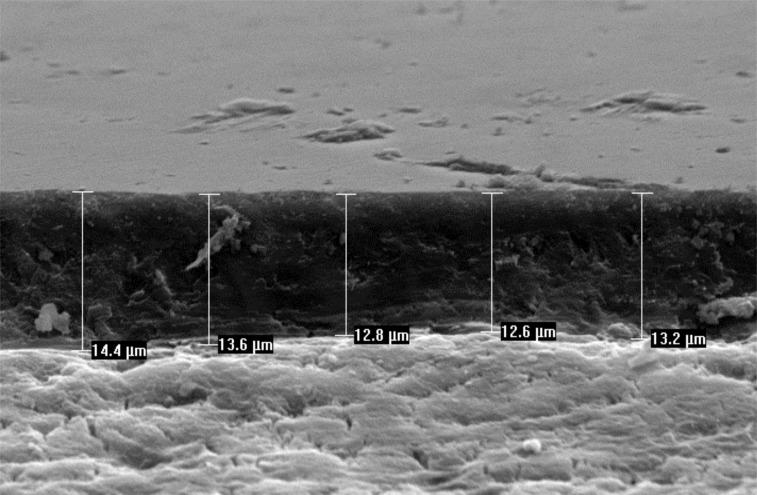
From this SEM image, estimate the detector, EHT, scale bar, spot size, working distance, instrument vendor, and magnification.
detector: SE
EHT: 20 kV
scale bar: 20000 nm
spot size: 4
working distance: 10.5 mm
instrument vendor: FEI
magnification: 1.4 K X